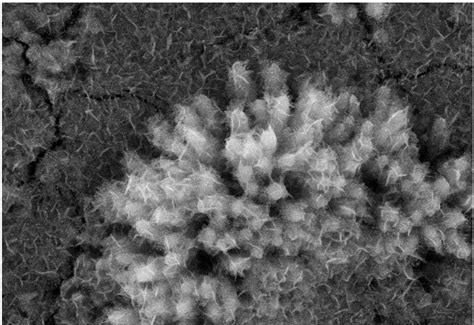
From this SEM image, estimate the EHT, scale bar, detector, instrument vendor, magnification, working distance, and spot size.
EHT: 20 kV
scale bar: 2000 nm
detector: SEI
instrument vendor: JEOL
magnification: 10 K X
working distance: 13 mm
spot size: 30